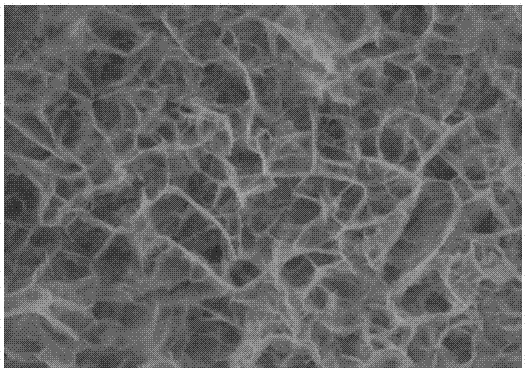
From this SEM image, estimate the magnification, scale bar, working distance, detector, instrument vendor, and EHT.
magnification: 100 K X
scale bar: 500 nm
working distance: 7.05 mm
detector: InBeam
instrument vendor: Tescan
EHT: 5 kV